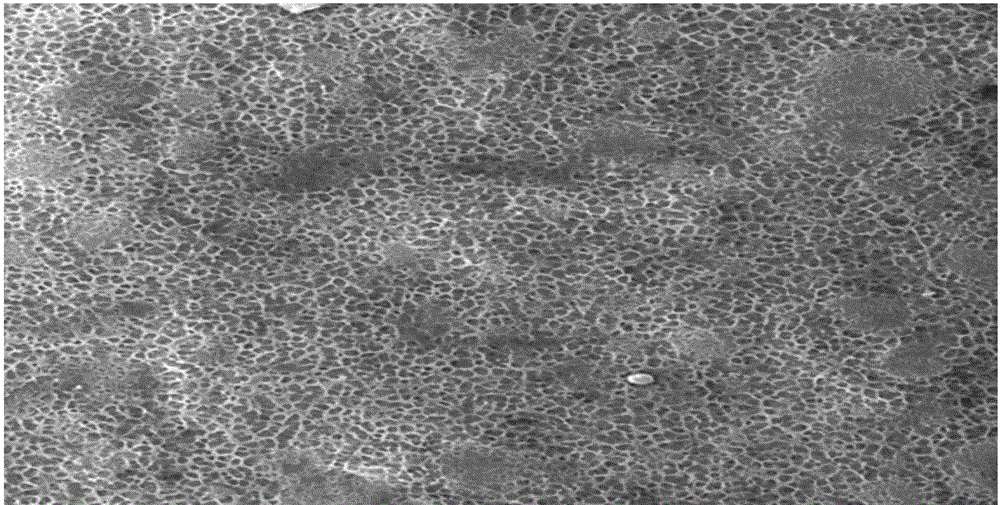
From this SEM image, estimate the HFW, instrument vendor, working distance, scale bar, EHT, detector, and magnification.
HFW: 224.4 µm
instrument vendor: Tescan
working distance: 15.19 mm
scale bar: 50000 nm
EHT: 20 kV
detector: SE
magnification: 1 K X